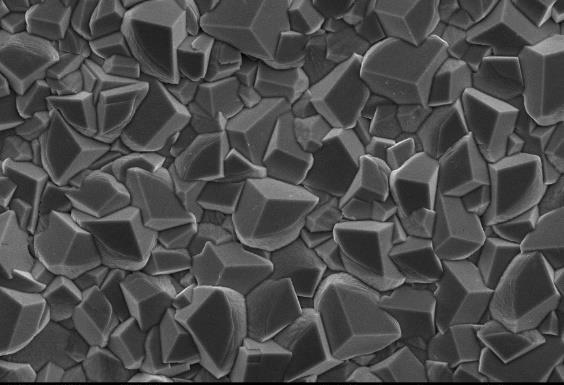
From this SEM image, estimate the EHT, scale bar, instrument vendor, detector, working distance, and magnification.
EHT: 15 kV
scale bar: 10000 nm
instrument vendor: JEOL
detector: SEI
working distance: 11 mm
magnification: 1 K X